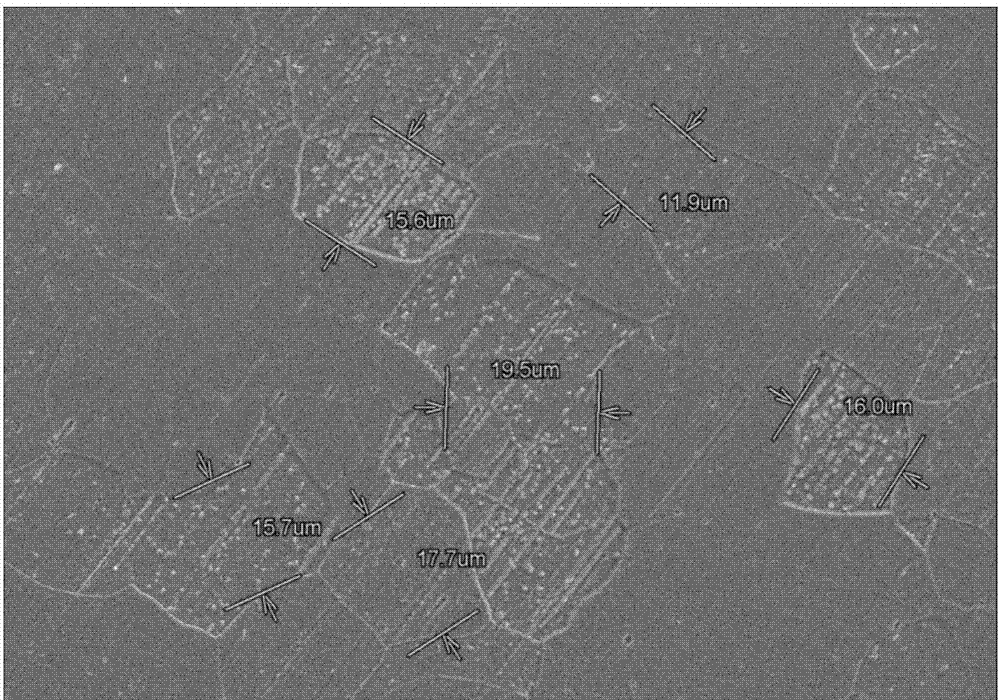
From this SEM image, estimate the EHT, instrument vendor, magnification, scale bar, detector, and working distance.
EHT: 15 kV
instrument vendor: Hitachi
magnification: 1 K X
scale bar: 50000 nm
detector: SE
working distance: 26.1 mm